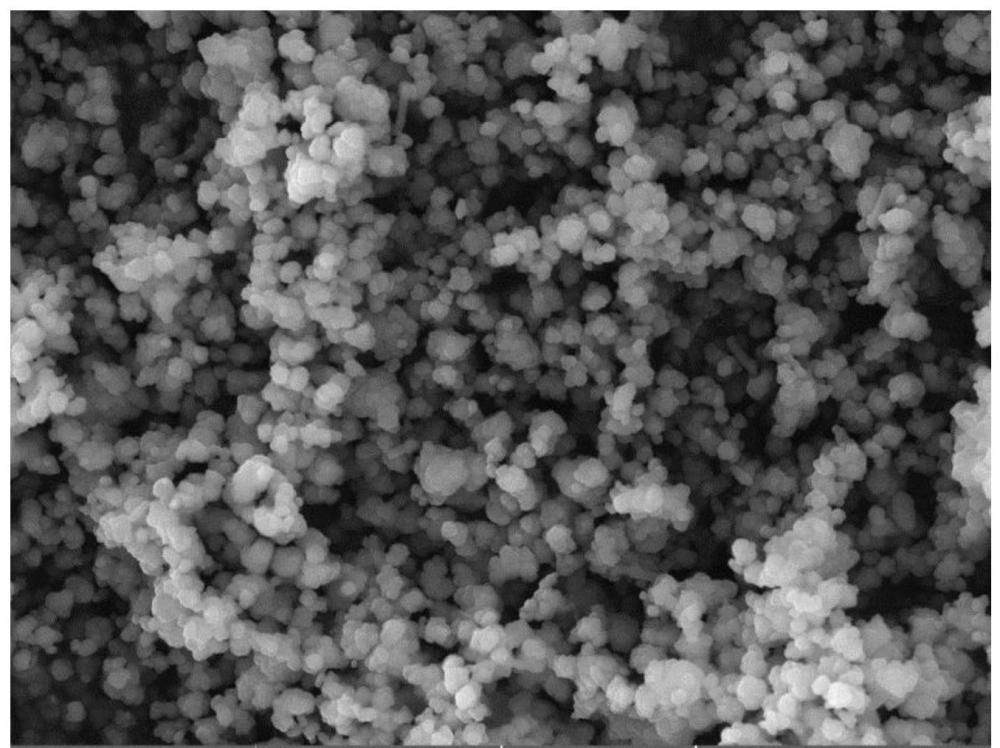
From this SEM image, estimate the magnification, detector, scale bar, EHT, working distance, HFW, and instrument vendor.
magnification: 10 K X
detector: SE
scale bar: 5000 nm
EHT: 15 kV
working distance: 10.38 mm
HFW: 25.3 µm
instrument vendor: Tescan